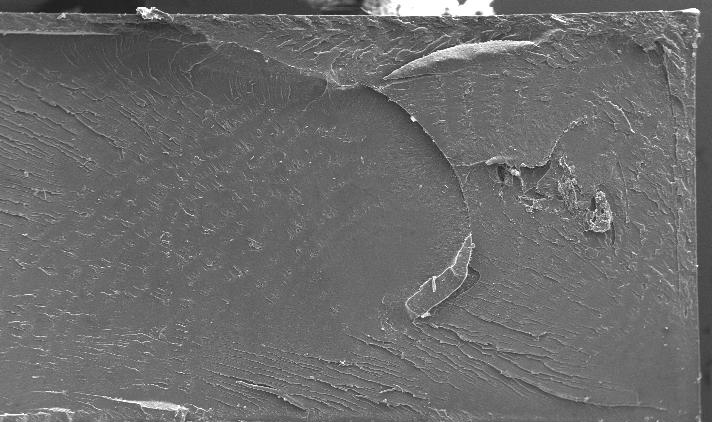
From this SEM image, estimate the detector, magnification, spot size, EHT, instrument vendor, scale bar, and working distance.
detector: SE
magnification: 0.05 K X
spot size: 5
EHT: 20 kV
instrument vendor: FEI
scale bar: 1000000 nm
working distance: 9.8 mm